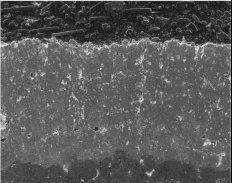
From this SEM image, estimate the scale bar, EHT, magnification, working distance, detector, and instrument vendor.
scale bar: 100000 nm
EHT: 20 kV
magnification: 0.3 K X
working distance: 17 mm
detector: SE1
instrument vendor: Zeiss